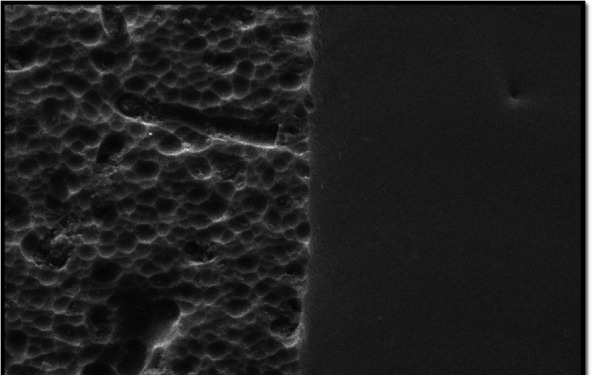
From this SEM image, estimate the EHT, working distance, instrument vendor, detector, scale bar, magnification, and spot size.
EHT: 20 kV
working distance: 9.4 mm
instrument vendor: FEI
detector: SE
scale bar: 10000 nm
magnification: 4 K X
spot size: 4